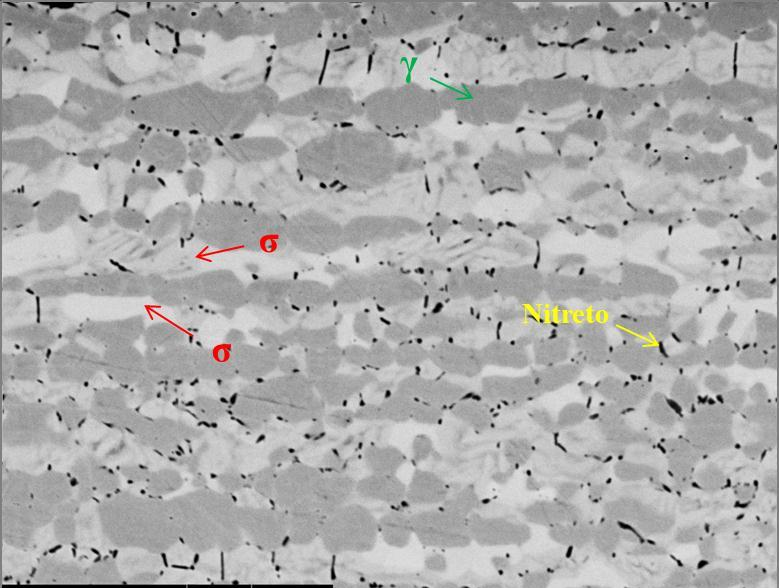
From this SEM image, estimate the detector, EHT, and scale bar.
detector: BSC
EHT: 20 kV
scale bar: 10000 nm